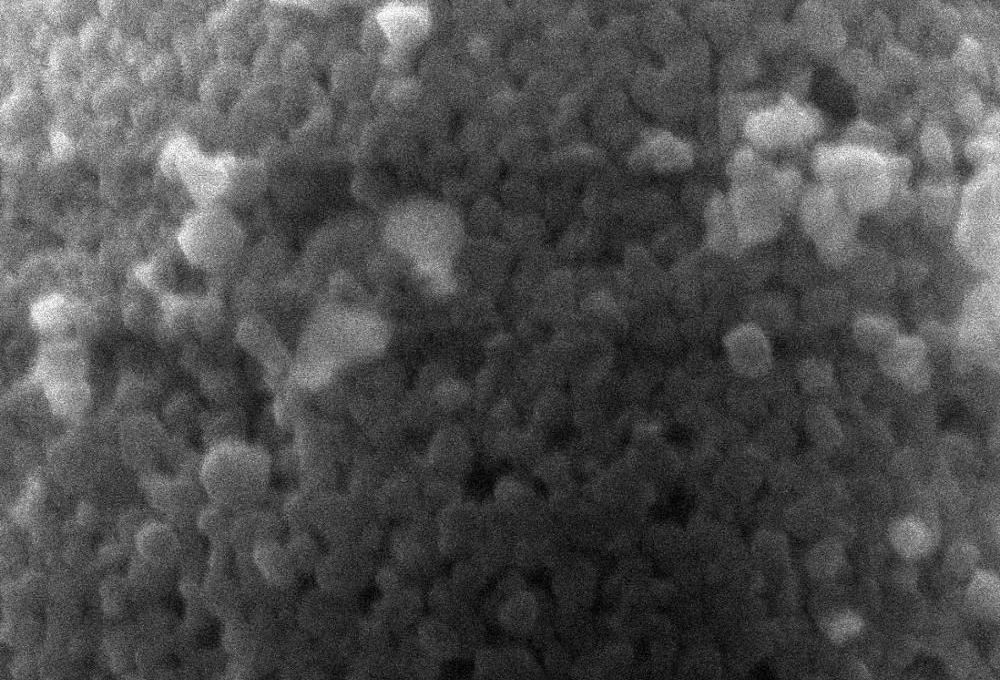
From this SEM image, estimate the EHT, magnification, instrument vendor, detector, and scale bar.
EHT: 20 kV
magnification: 30 K X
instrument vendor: JEOL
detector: SEI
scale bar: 500 nm